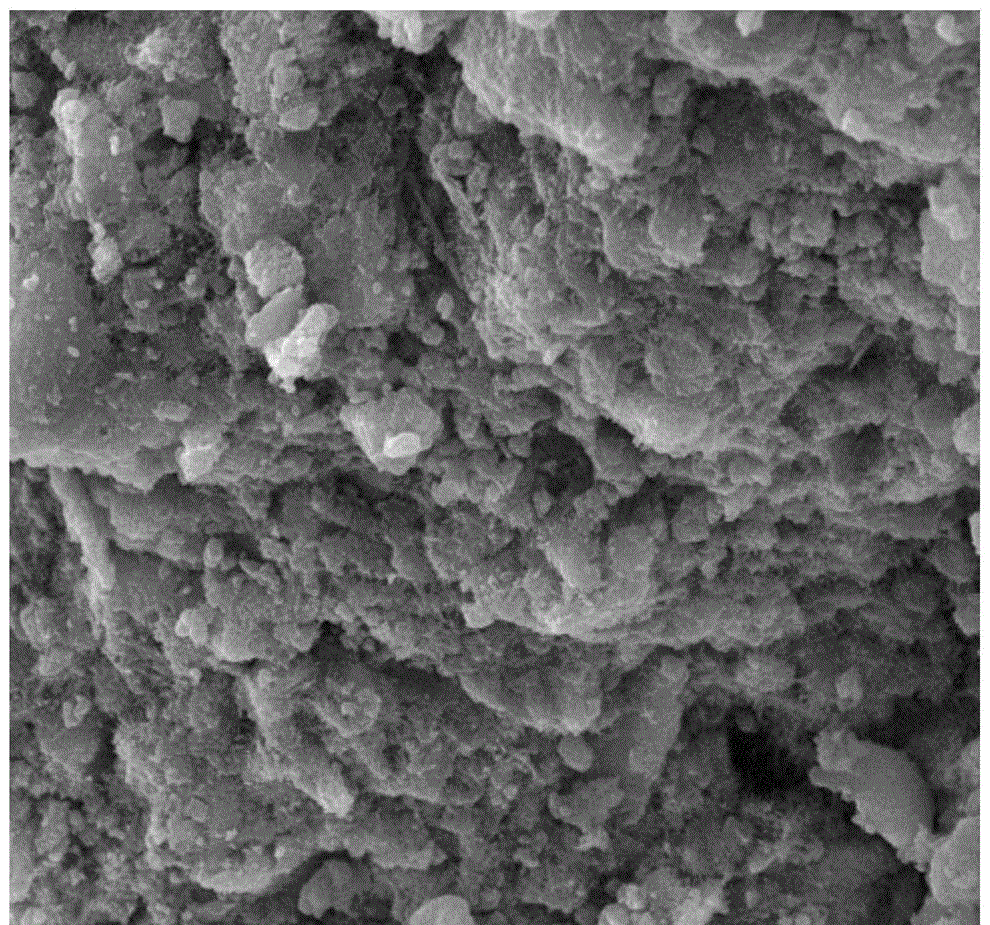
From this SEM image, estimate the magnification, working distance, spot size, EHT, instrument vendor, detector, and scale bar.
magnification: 5 K X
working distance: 16.4 mm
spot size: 3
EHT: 30 kV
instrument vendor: FEI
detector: ETD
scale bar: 20000 nm